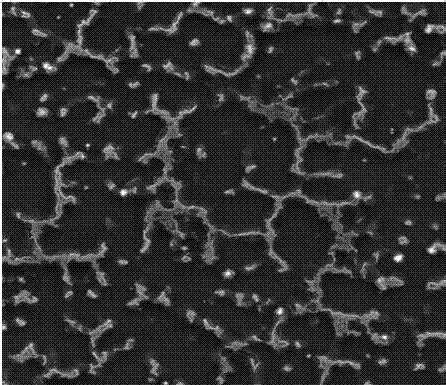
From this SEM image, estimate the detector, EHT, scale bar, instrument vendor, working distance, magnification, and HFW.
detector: SE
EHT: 30 kV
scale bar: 50000 nm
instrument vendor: Tescan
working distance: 14.65 mm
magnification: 1 K X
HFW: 208 µm